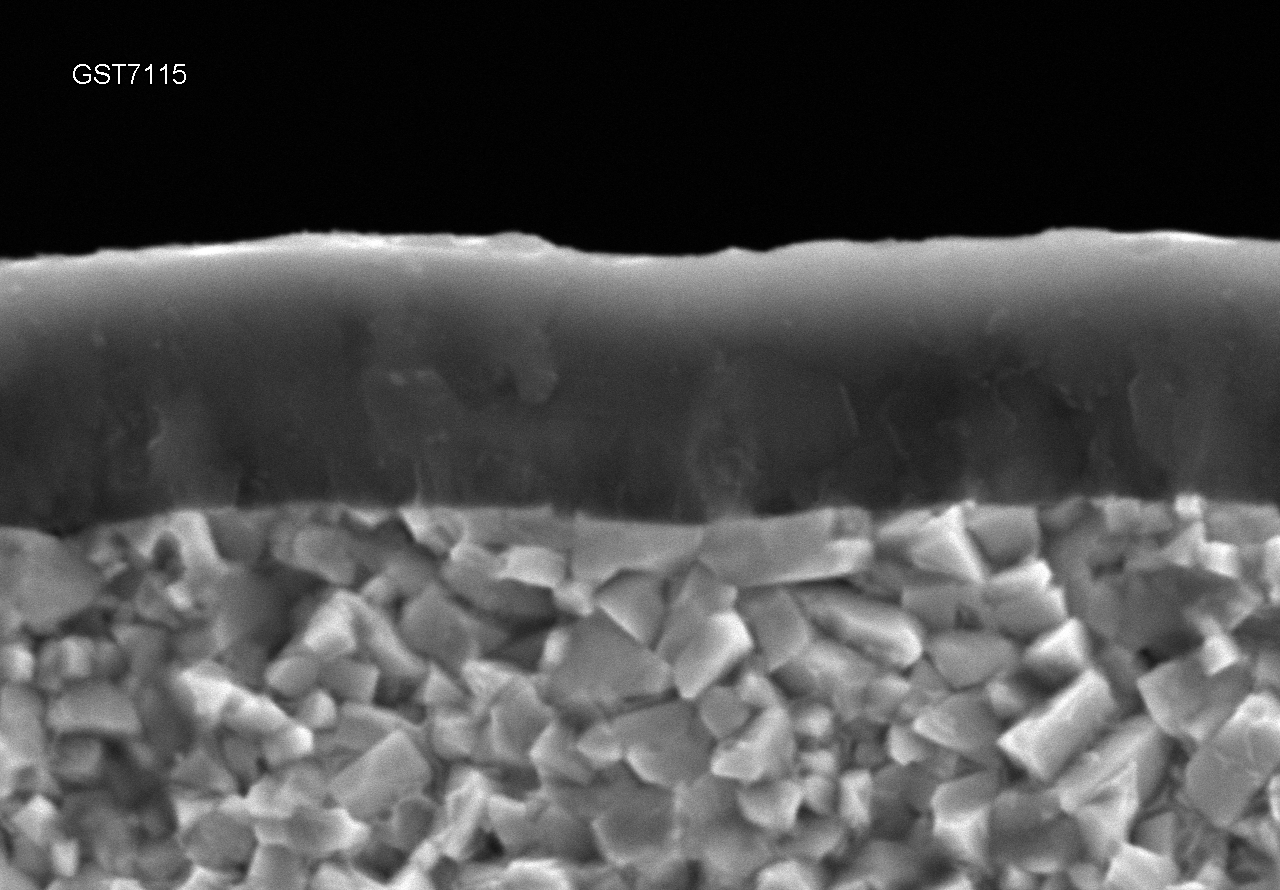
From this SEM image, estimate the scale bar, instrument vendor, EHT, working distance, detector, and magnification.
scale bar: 5000 nm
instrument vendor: Hitachi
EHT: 15 kV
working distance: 7.9 mm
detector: SE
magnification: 10 K X